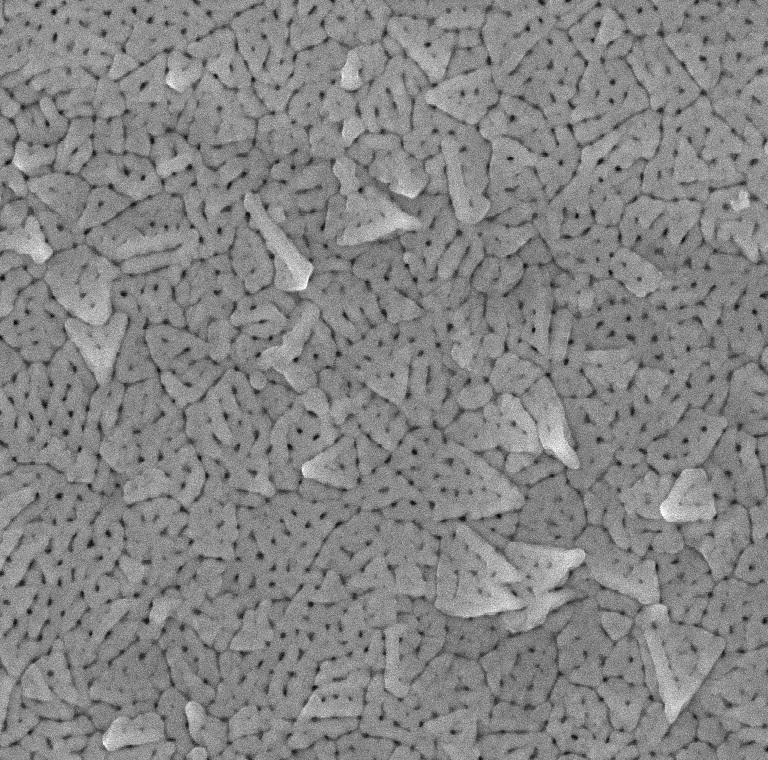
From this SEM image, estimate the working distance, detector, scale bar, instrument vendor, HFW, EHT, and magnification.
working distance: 3.469 mm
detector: InBeam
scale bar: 1000 nm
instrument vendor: Tescan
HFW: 4.011 µm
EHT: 15 kV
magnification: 54.02 K X